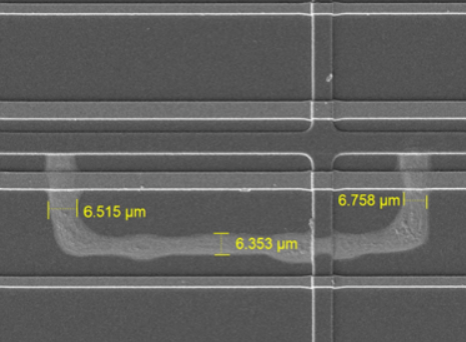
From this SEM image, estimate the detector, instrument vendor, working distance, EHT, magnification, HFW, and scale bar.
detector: SE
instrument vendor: Tescan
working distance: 9.99 mm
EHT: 10 kV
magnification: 2 K X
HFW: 138 µm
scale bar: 20000 nm